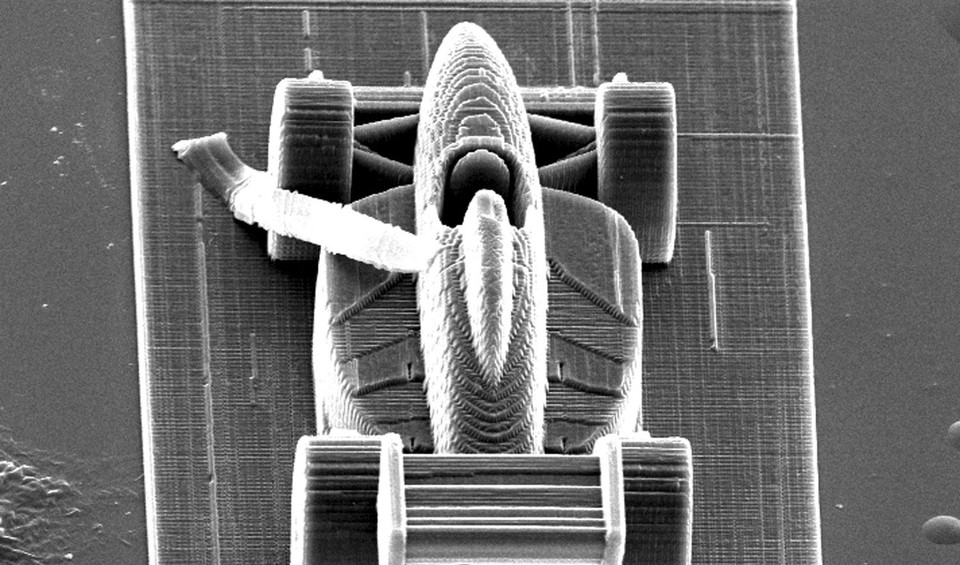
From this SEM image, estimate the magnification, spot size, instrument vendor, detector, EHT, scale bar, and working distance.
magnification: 0.404 K X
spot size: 4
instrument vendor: FEI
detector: SE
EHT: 20 kV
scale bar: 50000 nm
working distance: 27.2 mm